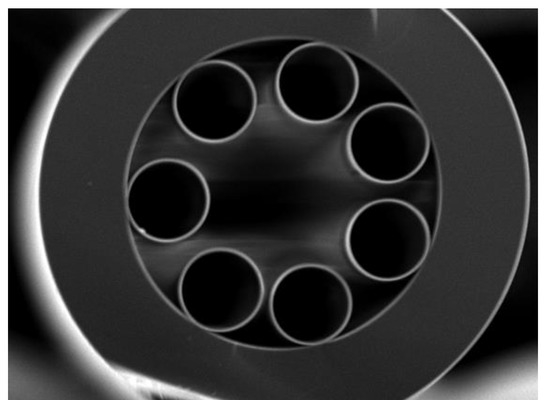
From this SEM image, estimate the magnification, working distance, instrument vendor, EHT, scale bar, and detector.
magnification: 0.35 K X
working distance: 11.1 mm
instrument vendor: JEOL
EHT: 15 kV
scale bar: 10000 nm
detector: SEI